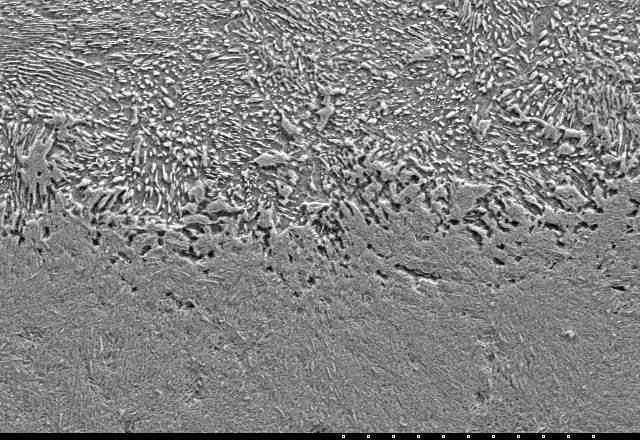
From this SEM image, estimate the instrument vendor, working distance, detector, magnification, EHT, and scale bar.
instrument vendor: Hitachi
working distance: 17.7 mm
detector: SE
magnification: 1 K X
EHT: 15 kV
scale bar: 50000 nm